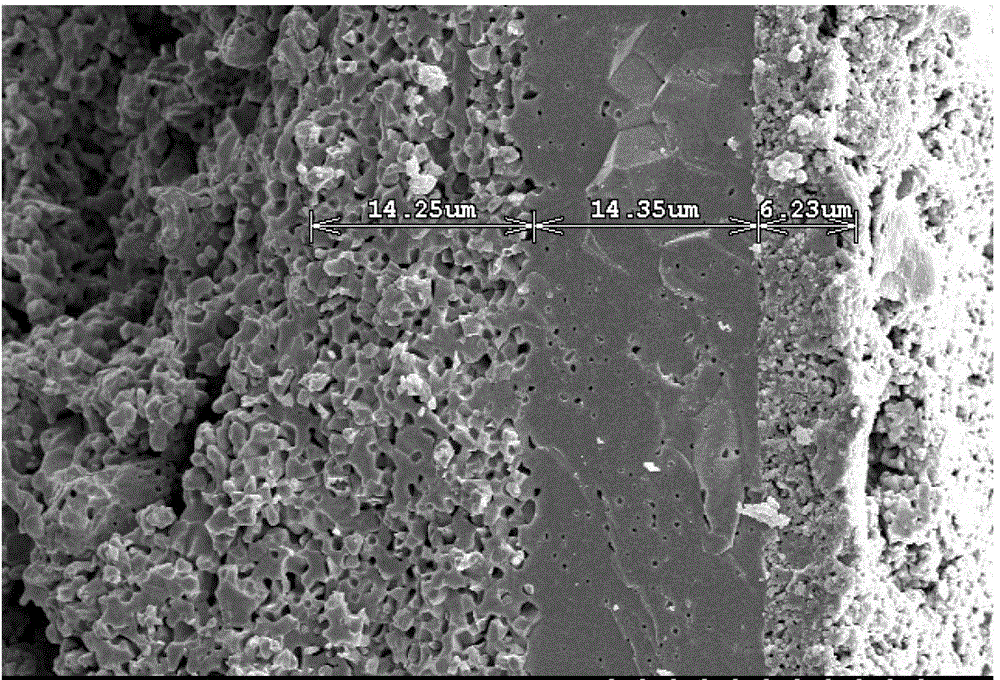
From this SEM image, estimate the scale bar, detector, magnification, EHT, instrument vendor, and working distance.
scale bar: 20000 nm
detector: SE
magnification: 2 K X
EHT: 10 kV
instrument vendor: Hitachi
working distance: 13.4 mm